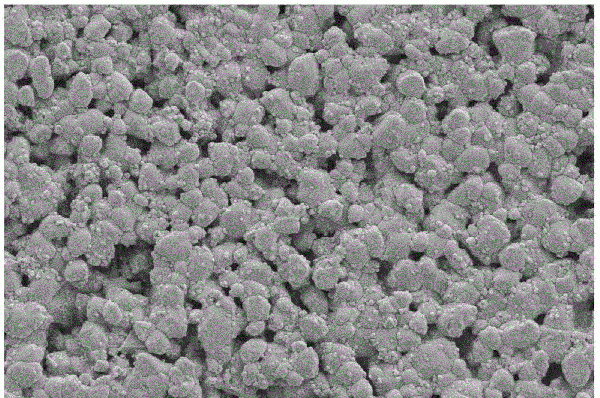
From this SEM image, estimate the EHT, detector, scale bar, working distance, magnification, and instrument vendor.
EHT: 5 kV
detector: SE2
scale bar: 1000 nm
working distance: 8.1 mm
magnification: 5 K X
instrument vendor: Zeiss